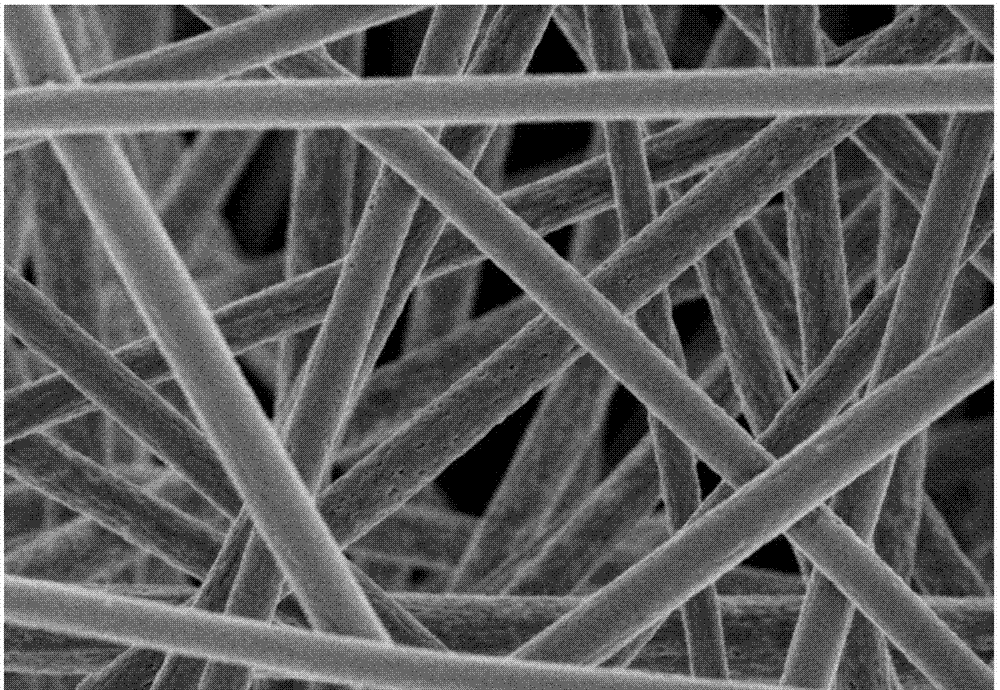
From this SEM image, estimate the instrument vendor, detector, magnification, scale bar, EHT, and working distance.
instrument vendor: Hitachi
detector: SE(M)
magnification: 5 K X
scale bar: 10000 nm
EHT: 3 kV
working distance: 9.1 mm